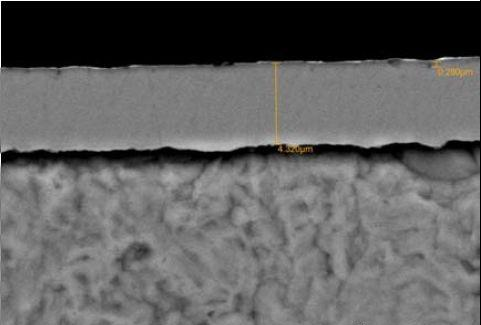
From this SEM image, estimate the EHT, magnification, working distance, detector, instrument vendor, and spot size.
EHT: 20 kV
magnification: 5 K X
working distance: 13 mm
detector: BEC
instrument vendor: JEOL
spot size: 50